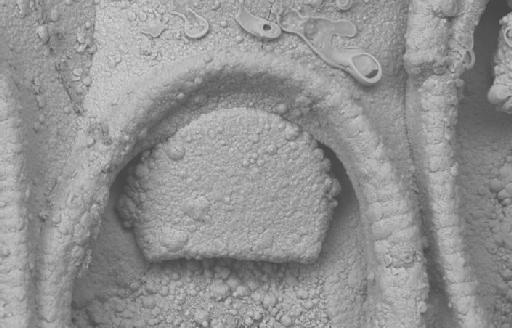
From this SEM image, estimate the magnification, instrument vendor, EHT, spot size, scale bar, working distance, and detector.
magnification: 1 K X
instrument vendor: Zeiss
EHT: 15 kV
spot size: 500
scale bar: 20000 nm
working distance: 17 mm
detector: QBSD